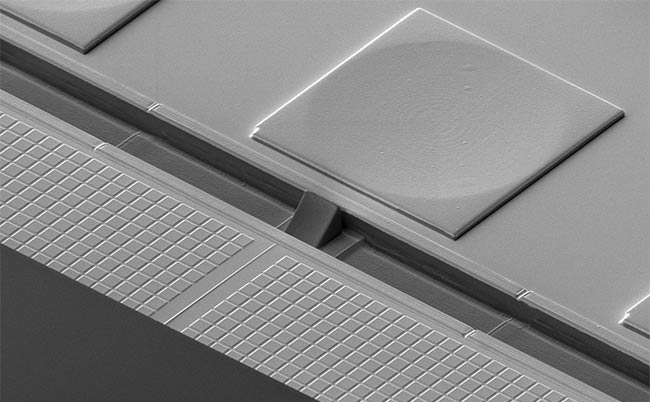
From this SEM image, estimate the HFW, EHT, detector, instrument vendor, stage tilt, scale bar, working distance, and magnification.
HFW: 345 µm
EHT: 2 kV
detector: ICE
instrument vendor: FEI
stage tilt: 52°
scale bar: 100000 nm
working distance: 3.7 mm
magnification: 1.2 K X